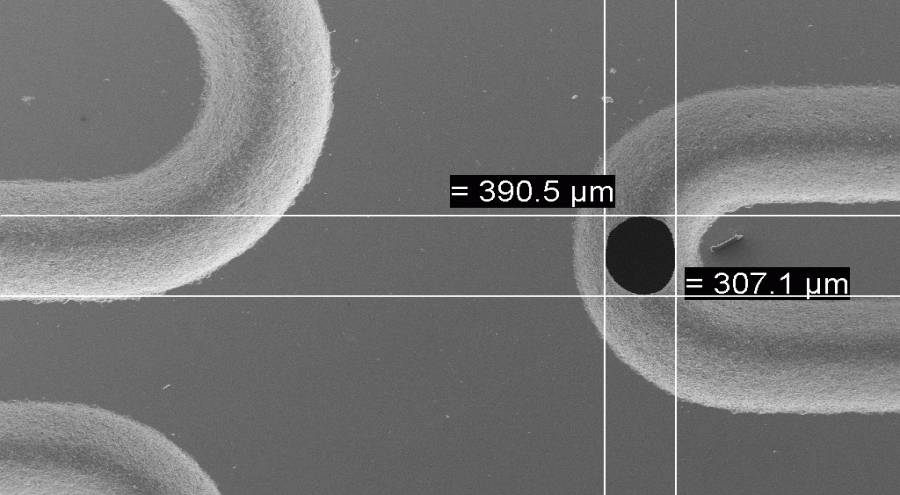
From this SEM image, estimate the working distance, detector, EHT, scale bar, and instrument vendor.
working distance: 13 mm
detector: SE1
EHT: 20 kV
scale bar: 300000 nm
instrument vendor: Zeiss